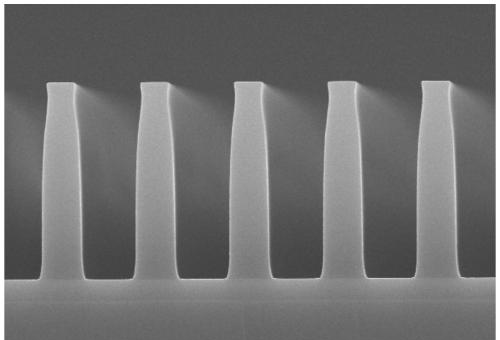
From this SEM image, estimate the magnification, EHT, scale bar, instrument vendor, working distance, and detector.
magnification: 6 K X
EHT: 20 kV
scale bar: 5000 nm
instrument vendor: Hitachi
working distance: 9.8 mm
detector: SE(M)